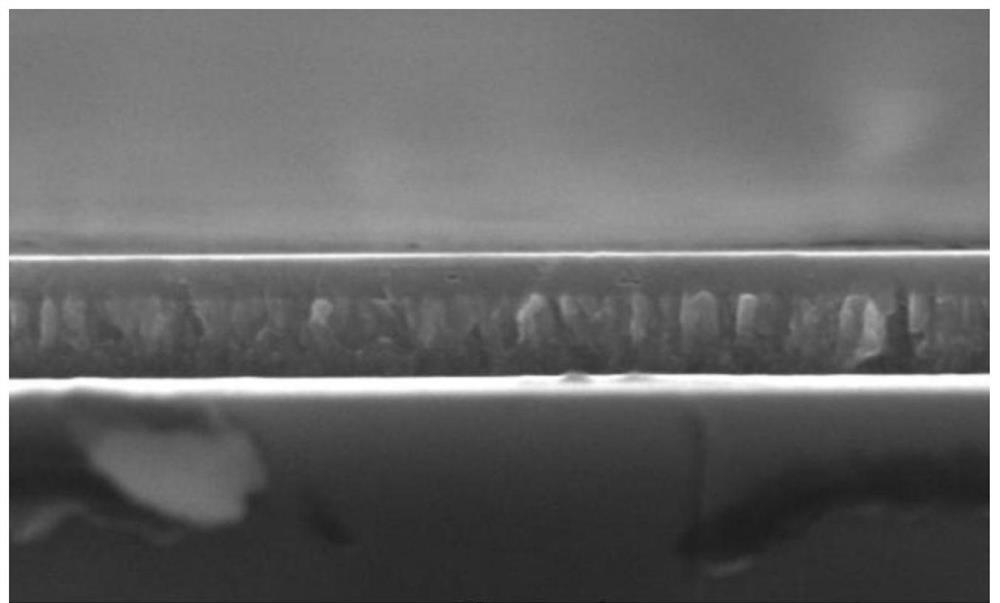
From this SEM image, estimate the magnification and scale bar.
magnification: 200 K X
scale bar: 500 nm